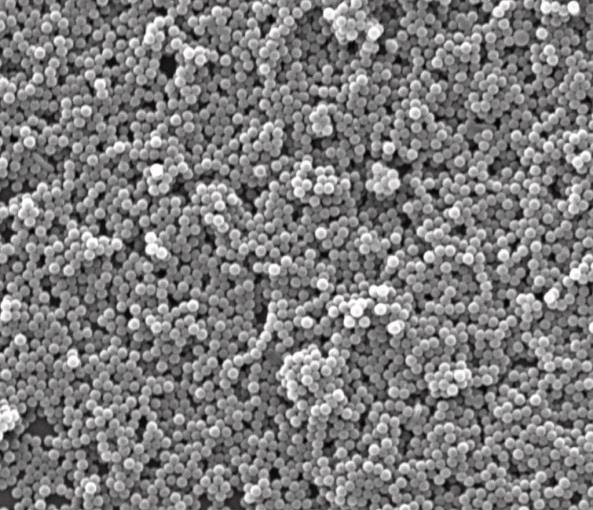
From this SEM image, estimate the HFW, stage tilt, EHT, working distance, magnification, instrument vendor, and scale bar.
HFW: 13.5 µm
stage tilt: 0°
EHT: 25 kV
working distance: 10 mm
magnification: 20 K X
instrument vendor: FEI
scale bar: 5000 nm